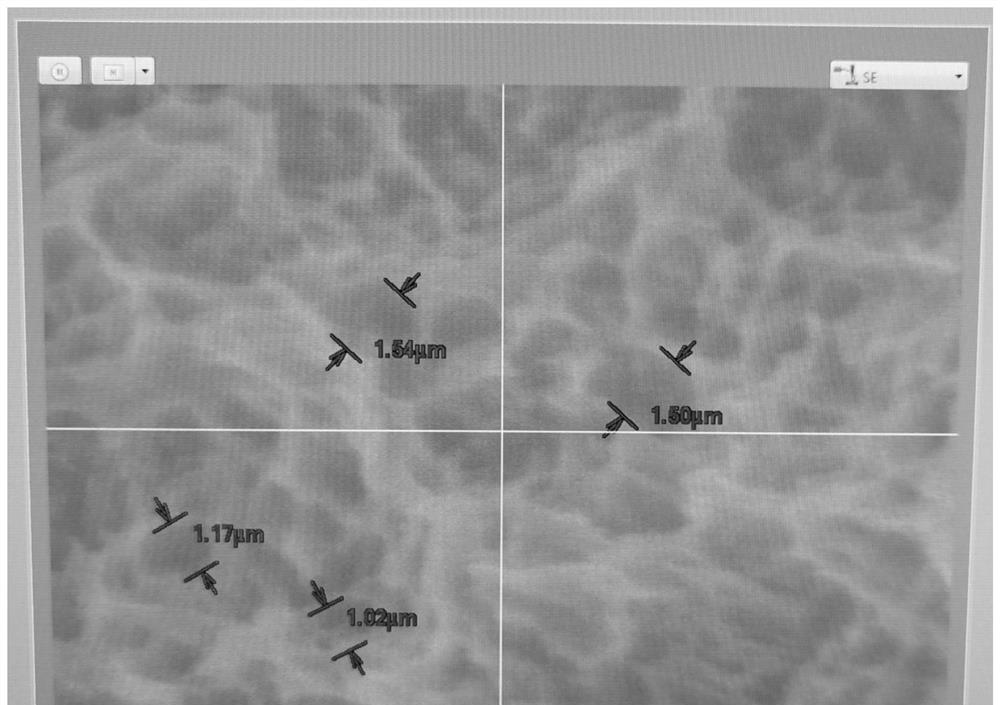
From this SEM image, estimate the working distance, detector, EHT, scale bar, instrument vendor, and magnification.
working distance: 9.1 mm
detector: SE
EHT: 10 kV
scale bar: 5000 nm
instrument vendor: Hitachi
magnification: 7.02 K X